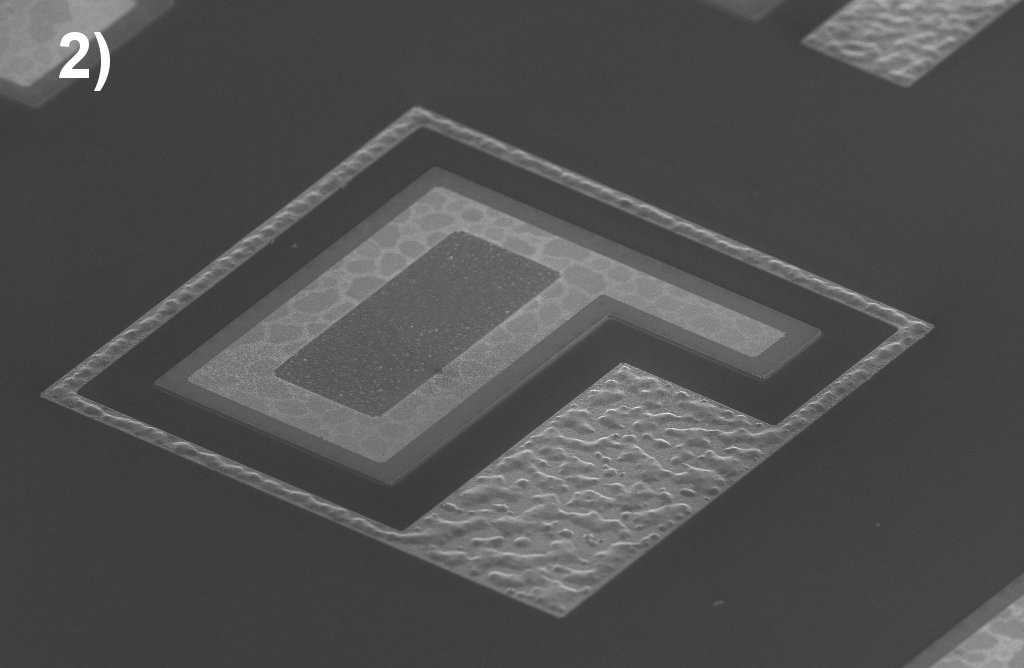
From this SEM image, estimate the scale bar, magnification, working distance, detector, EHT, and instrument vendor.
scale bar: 20000 nm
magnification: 0.3 K X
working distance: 6.5 mm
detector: InLens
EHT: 5 kV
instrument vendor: Zeiss